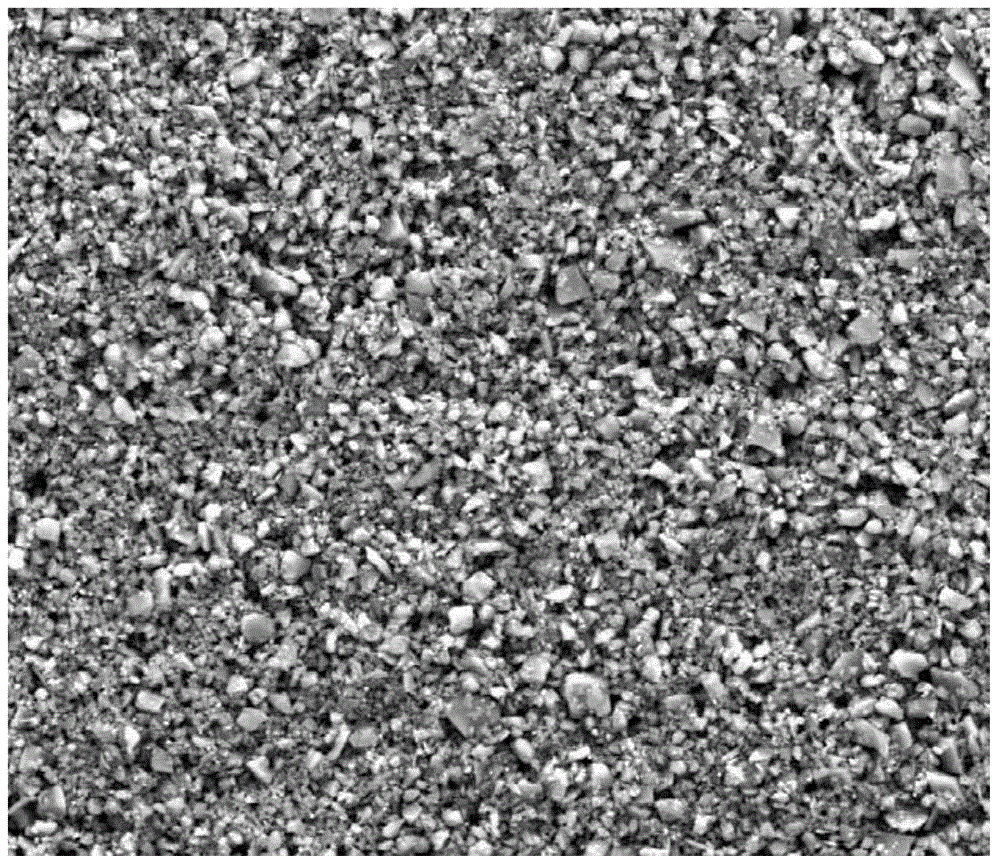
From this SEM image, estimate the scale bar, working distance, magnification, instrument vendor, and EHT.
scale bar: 50000 nm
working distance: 17.1 mm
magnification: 2 K X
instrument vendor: FEI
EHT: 20 kV